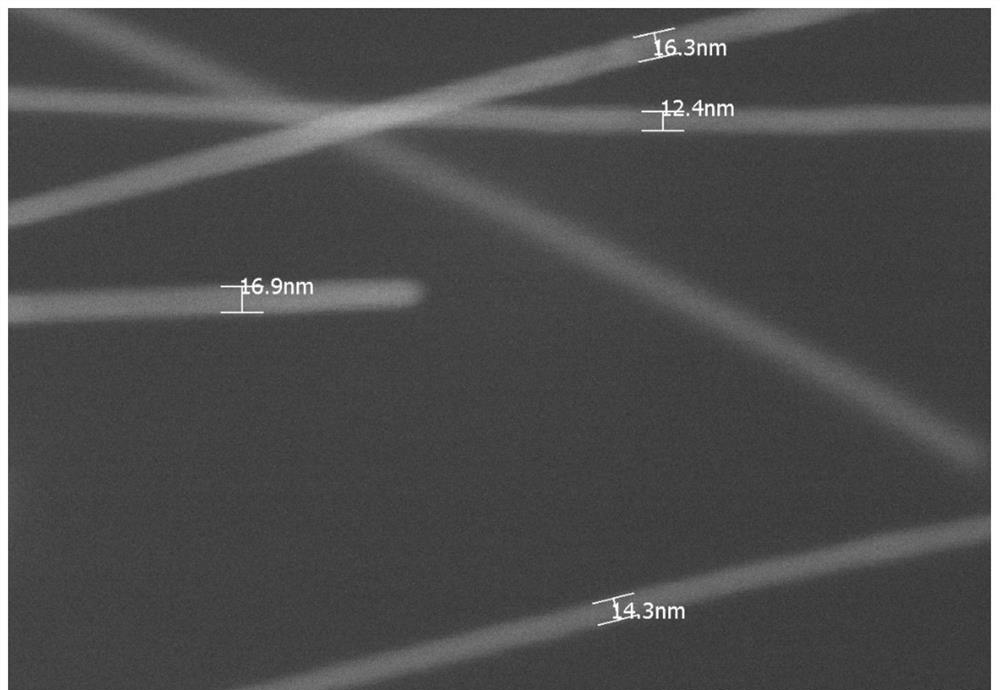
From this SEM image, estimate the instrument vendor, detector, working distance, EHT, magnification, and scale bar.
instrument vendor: Hitachi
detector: SE(L)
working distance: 9.3 mm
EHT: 15 kV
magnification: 200 K X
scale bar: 200 nm